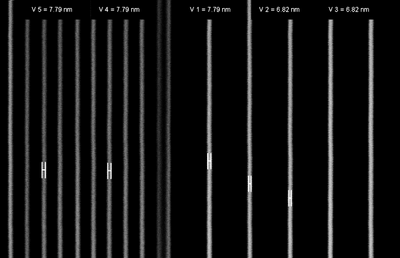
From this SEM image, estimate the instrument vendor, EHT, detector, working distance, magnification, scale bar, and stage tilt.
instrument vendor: Zeiss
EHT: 3 kV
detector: InLens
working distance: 4.8 mm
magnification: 301.68 K X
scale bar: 100 nm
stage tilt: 0°